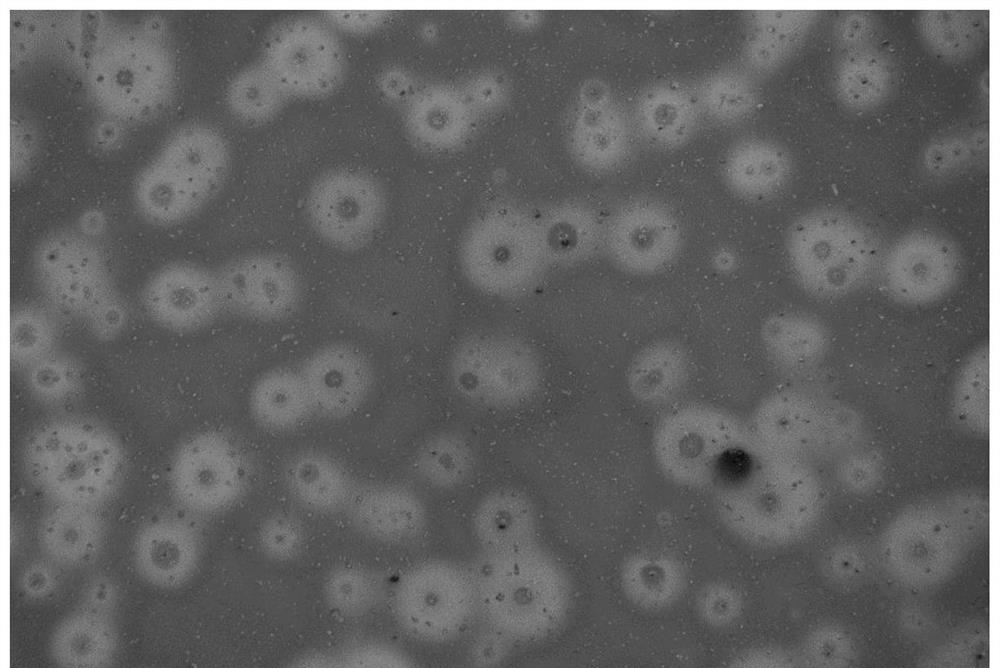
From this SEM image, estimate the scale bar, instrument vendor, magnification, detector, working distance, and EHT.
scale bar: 100000 nm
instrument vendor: Zeiss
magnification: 0.05 K X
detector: InLens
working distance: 8.7 mm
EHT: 5 kV